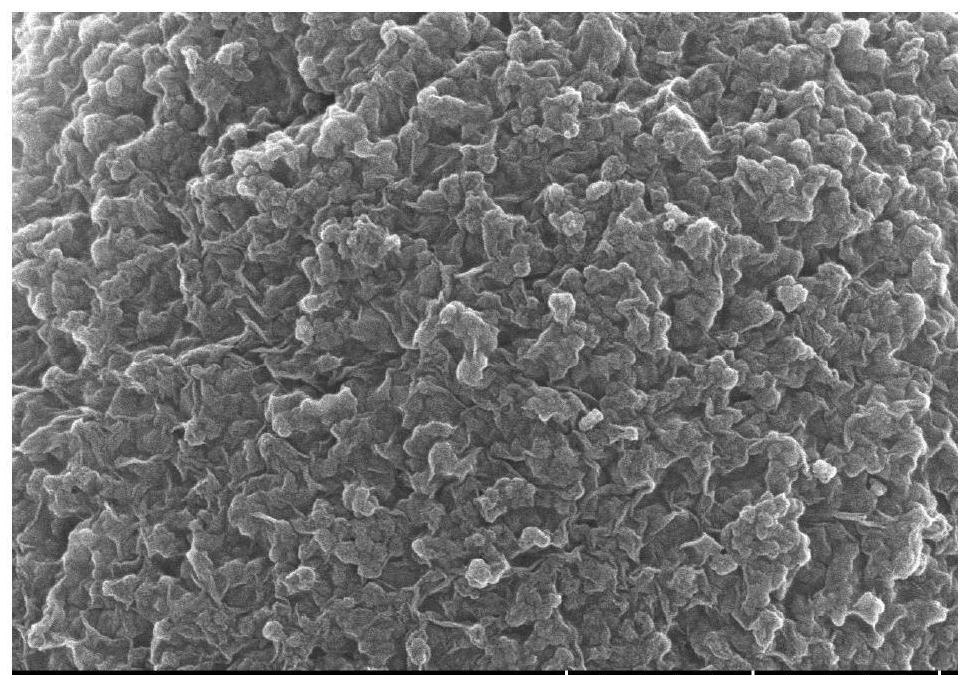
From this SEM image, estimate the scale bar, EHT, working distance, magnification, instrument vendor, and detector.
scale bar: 1000 nm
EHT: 5 kV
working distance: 4.8 mm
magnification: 50 K X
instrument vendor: Hitachi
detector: SE(UL)